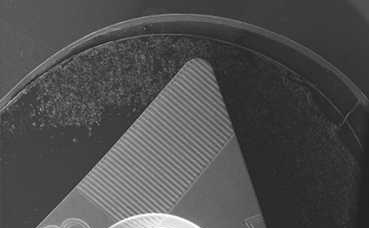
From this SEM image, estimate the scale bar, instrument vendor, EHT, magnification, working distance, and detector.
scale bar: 1000000 nm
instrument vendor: Zeiss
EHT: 20 kV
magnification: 0.013 K X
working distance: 21 mm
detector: SE1